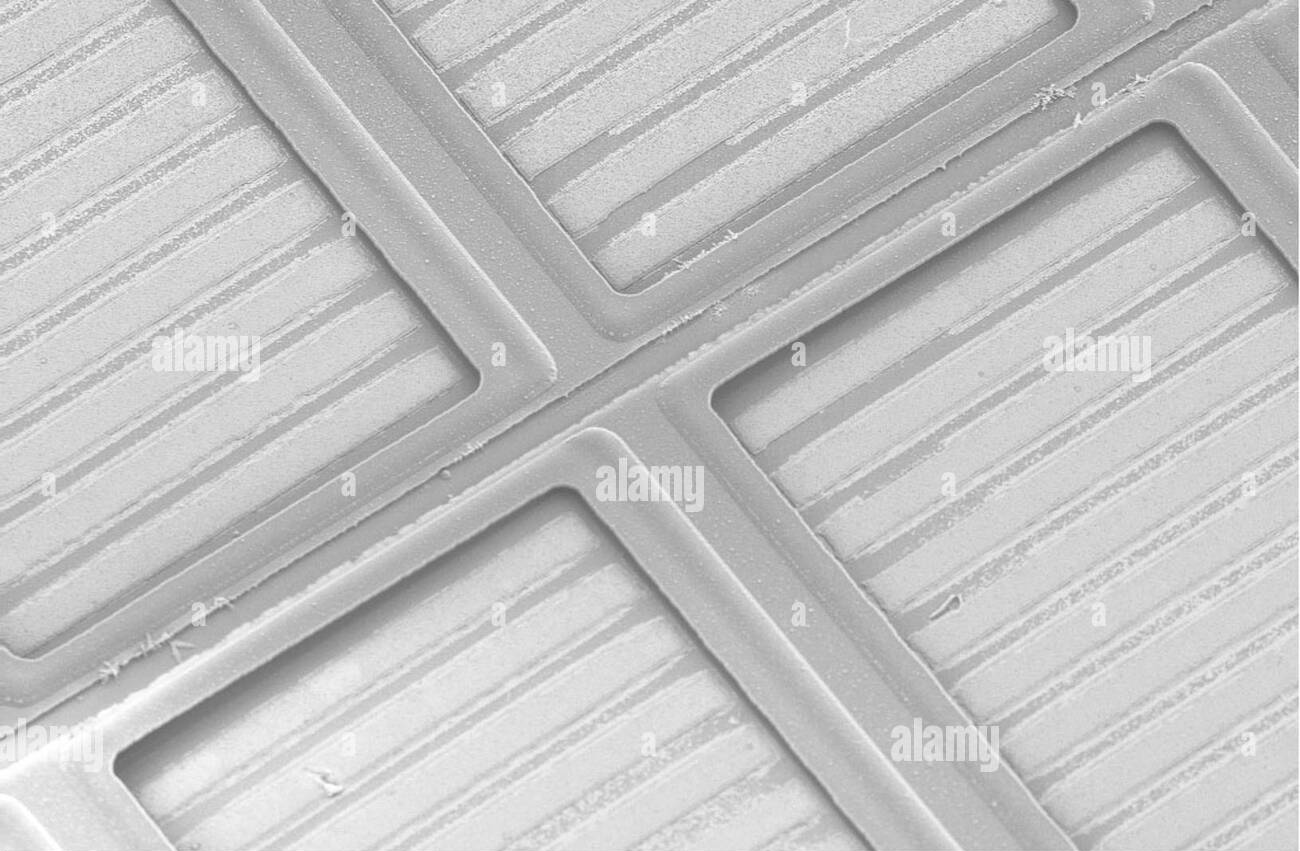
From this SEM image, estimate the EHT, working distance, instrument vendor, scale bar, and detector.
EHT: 5 kV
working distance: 10 mm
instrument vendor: Zeiss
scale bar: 20000 nm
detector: SE2